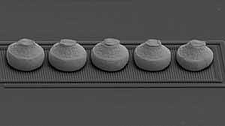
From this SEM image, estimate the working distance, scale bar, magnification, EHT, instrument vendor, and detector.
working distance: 27.9 mm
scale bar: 200000 nm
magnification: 0.25 K X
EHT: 20 kV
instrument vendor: JEOL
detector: SE